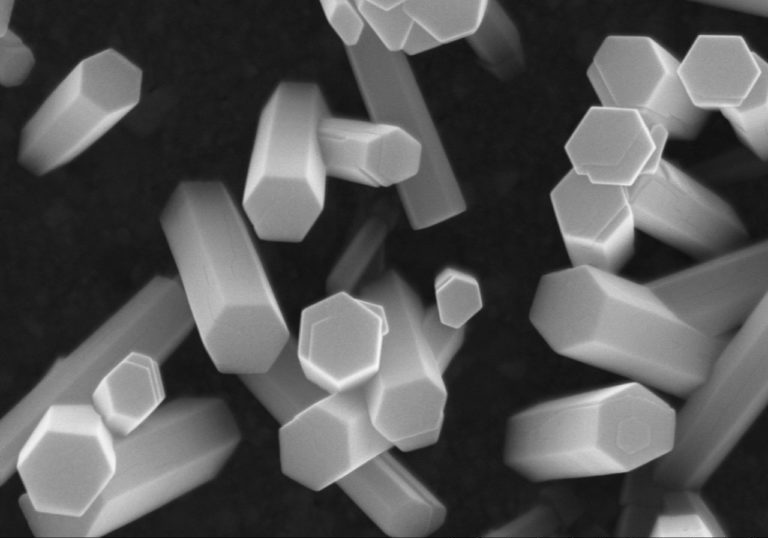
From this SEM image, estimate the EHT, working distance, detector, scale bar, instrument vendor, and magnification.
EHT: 15 kV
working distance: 6.4 mm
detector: SE(M)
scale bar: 1000 nm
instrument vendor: Hitachi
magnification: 50 K X